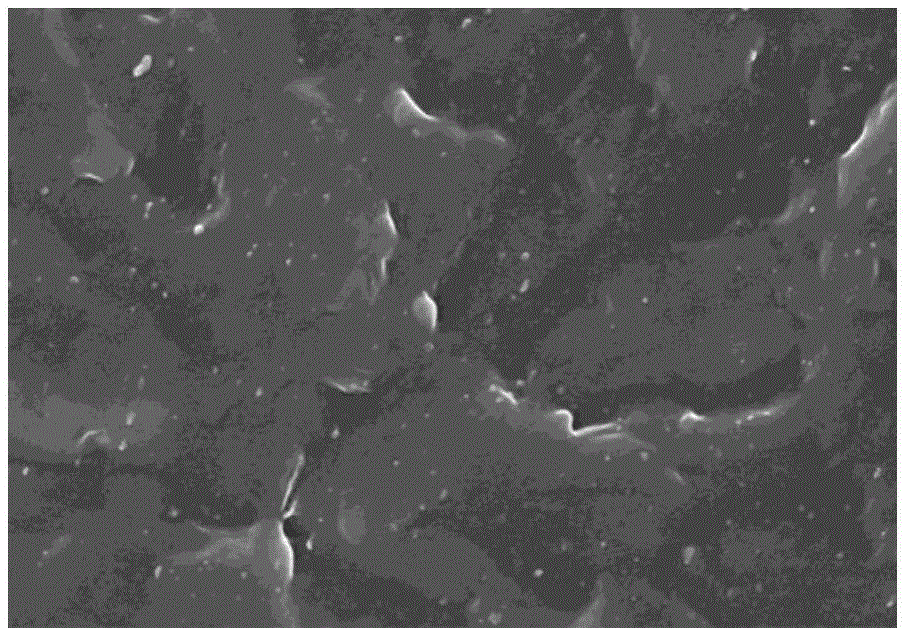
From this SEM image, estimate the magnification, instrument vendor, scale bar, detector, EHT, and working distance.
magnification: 5 K X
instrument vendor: Hitachi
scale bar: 10000 nm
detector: SE(M)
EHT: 10 kV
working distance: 7.4 mm